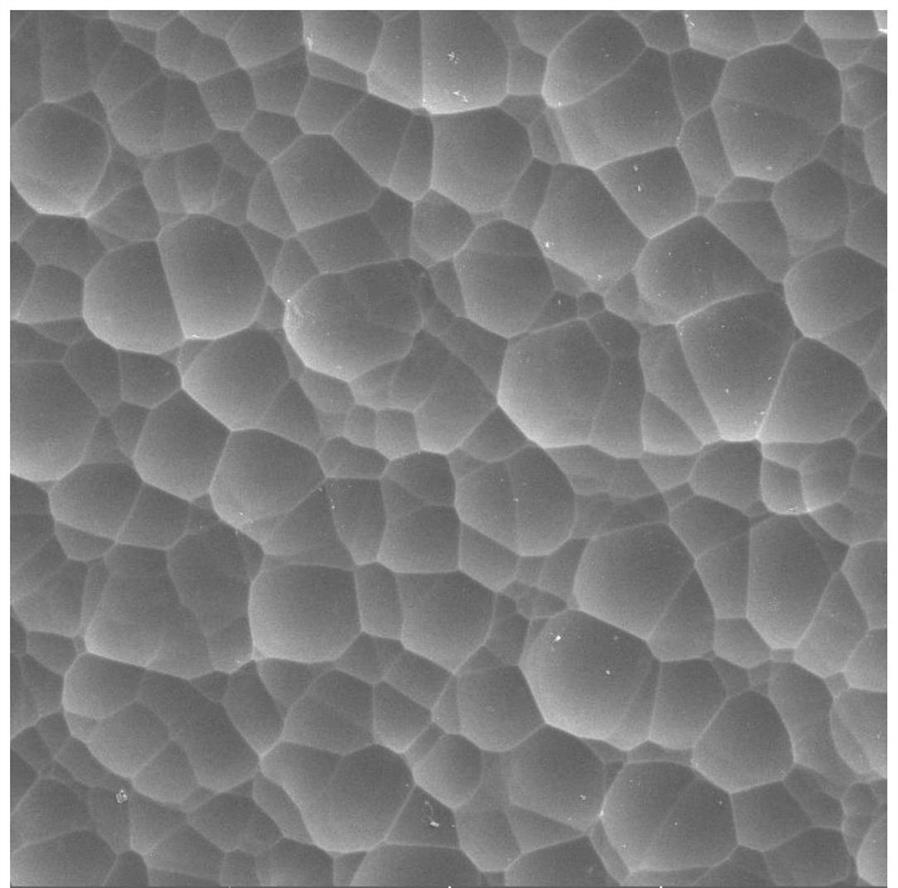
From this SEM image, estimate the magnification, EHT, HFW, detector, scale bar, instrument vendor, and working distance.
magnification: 5 K X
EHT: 5 kV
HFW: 41.5 µm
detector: SE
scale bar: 10000 nm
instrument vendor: Tescan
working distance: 10.15 mm